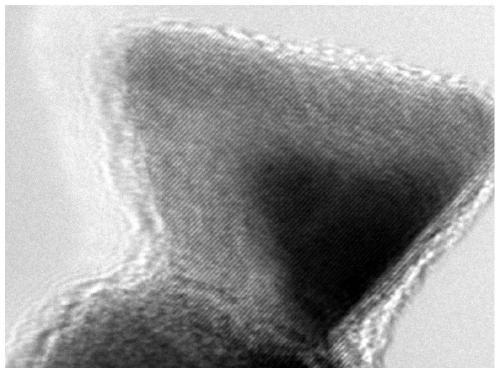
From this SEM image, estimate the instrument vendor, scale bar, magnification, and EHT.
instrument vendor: JEOL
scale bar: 5 nm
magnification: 800 K X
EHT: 200 kV